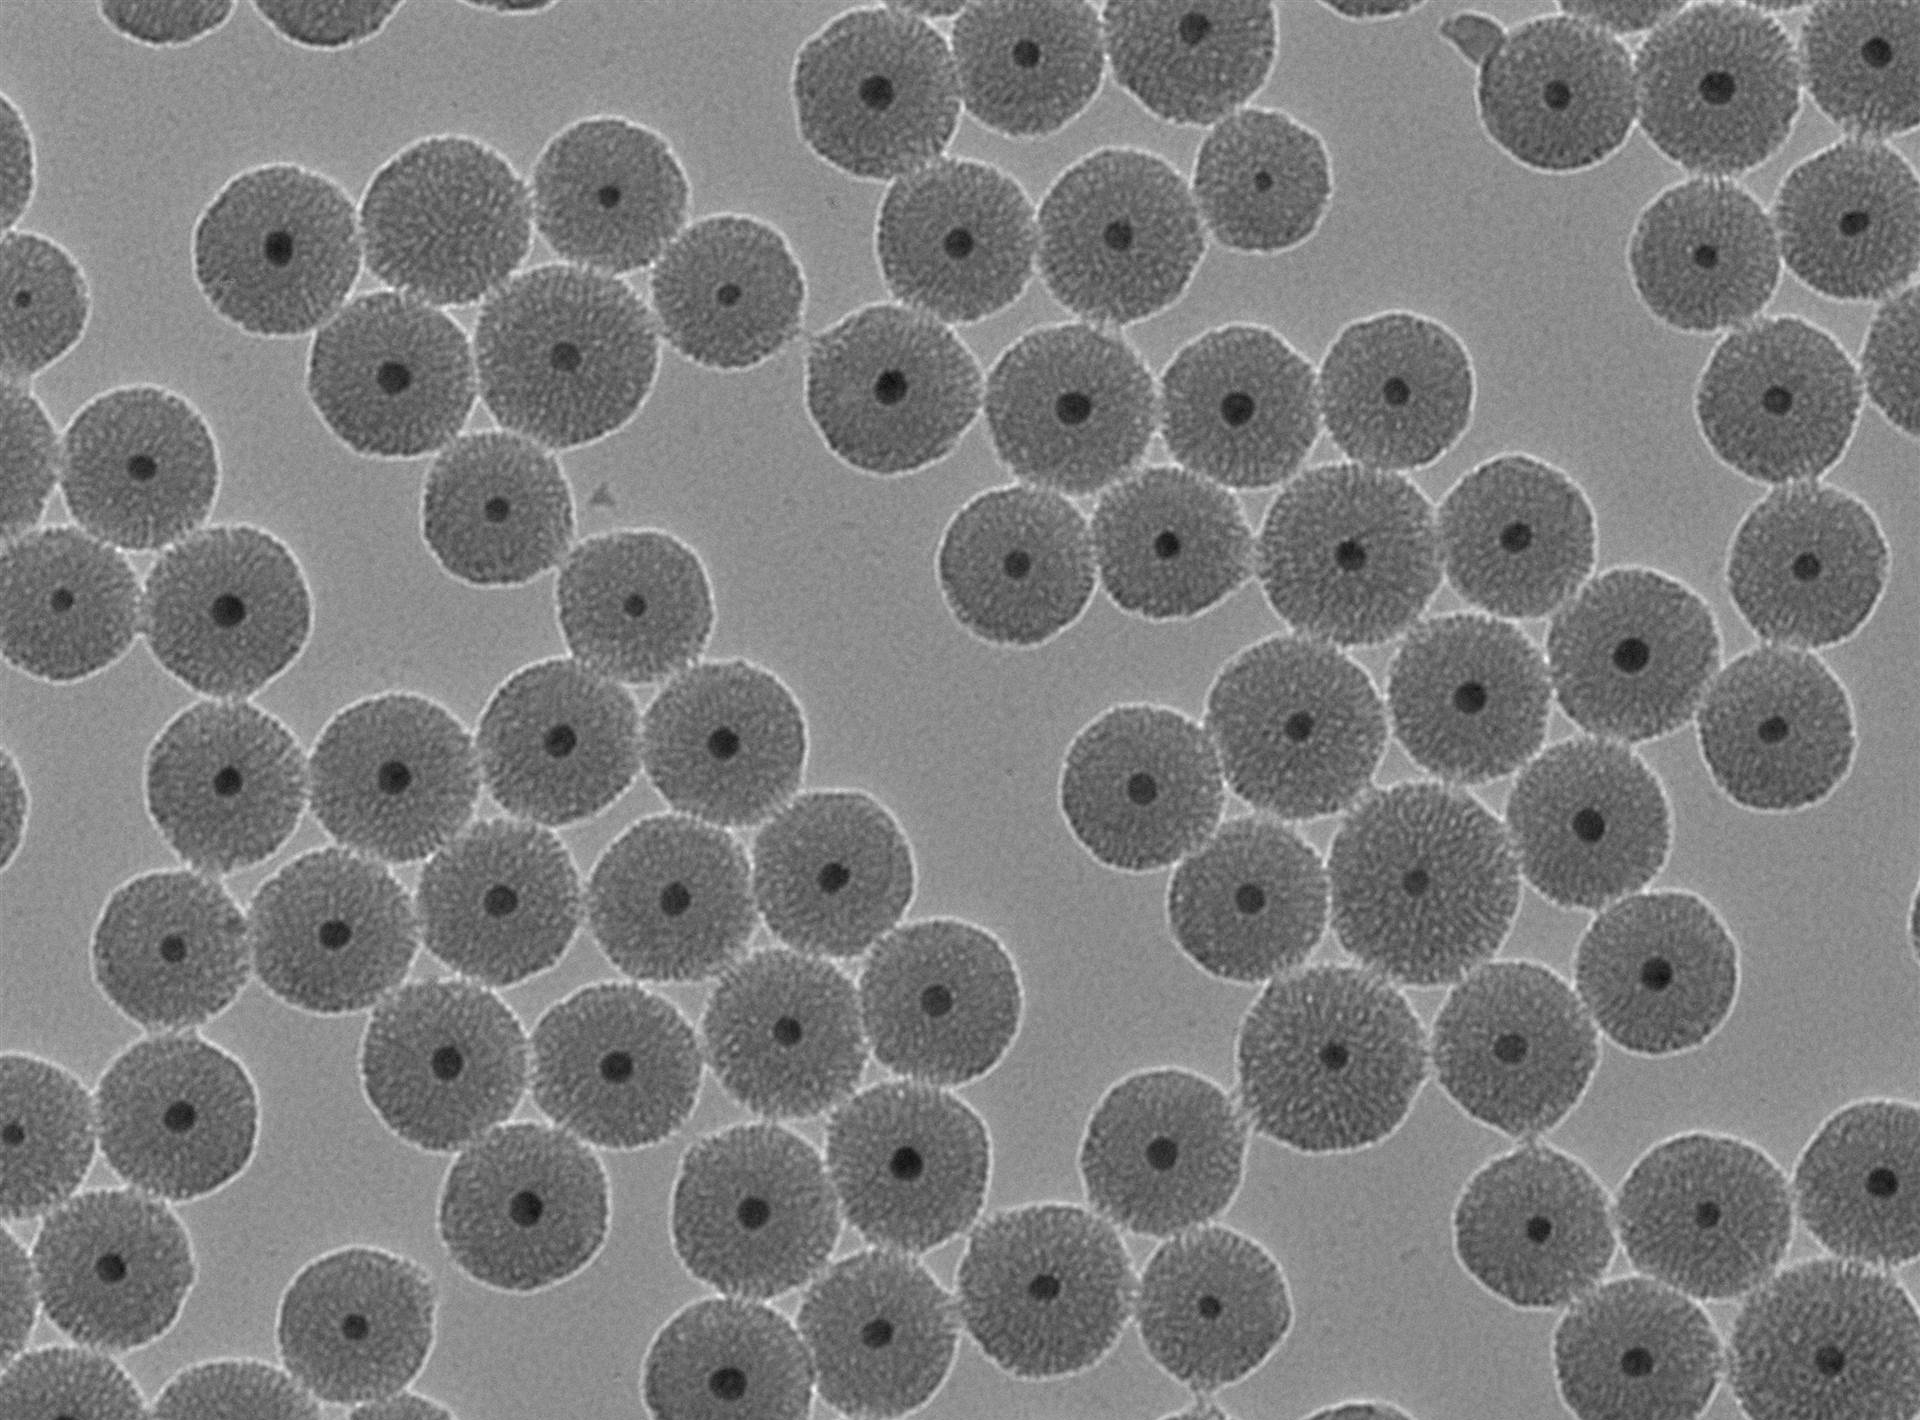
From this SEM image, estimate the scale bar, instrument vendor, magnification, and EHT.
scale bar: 200 nm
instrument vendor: JEOL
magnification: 40 K X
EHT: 100 kV